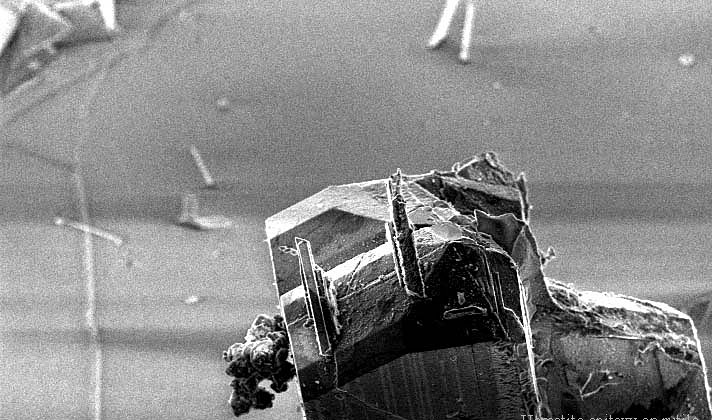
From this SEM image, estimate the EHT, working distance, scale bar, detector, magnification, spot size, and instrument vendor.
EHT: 10 kV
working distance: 6.3 mm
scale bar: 50000 nm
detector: SE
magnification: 0.4 K X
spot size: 3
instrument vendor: FEI